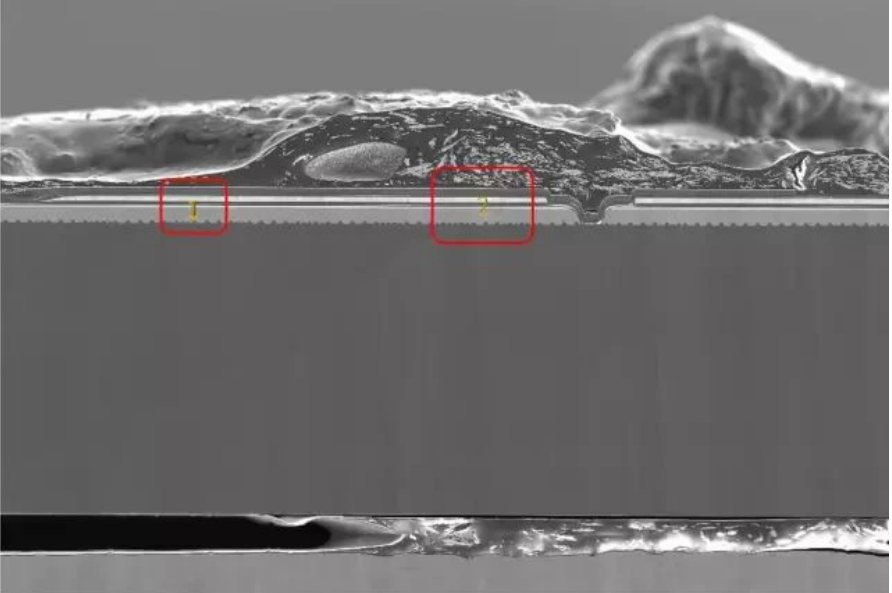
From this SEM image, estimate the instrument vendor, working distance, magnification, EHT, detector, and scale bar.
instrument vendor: Zeiss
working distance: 6.2 mm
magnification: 0.3 K X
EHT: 5 kV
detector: SE2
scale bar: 20000 nm